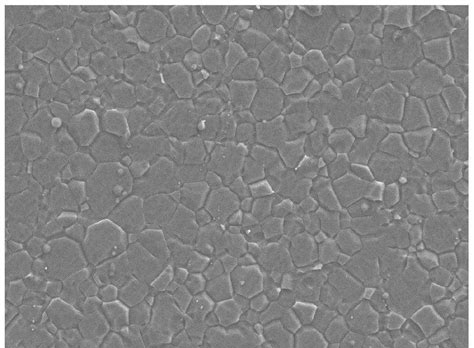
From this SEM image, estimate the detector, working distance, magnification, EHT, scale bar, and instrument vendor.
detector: LED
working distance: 9.6 mm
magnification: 1 K X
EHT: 10 kV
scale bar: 10000 nm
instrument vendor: JEOL